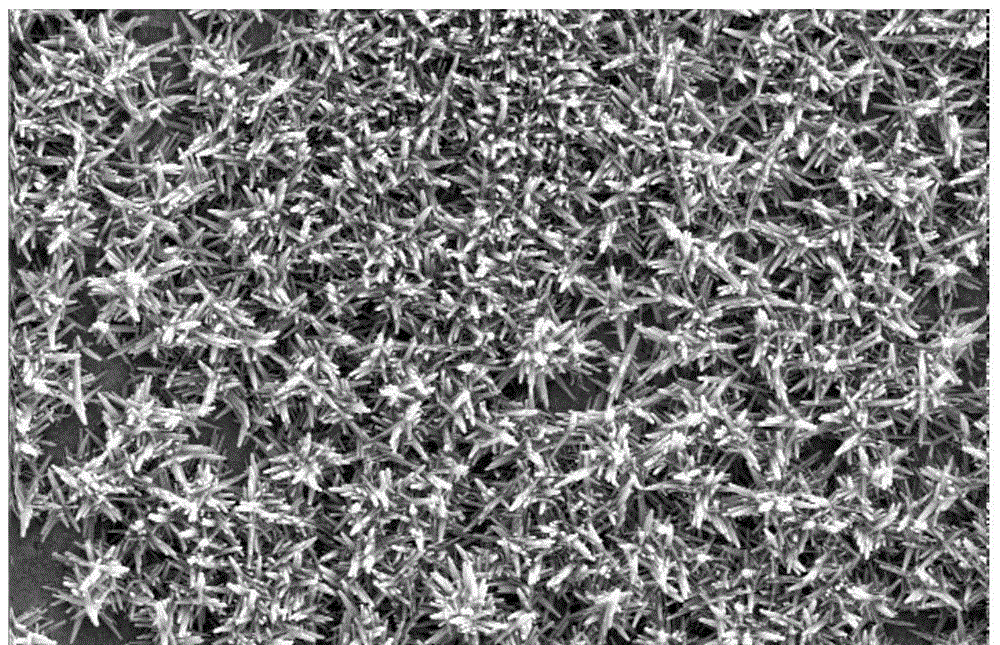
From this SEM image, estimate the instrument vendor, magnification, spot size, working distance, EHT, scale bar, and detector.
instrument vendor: FEI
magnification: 10 K X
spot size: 3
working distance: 5.5 mm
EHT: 20 kV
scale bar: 5000 nm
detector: SE